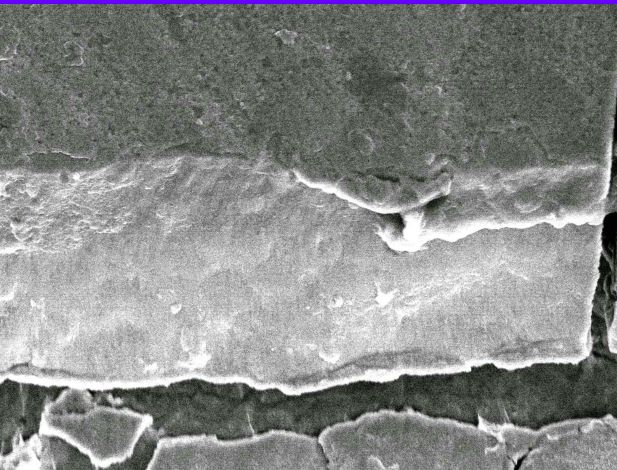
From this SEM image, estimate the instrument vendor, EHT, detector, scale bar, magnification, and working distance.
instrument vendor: JEOL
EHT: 12 kV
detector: SEI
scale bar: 1000 nm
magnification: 4 K X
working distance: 7.9 mm